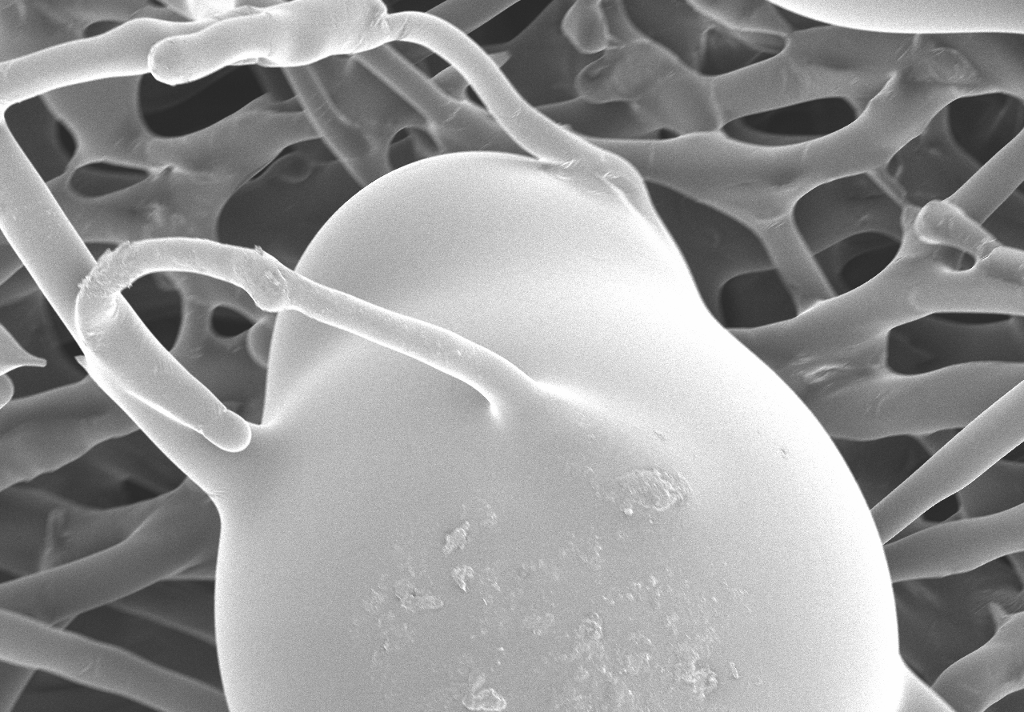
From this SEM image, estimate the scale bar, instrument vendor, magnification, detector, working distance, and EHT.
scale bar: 100000 nm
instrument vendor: Hitachi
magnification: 0.3 K X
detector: SE(M)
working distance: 11.3 mm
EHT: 3 kV